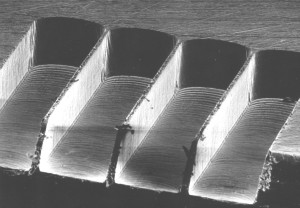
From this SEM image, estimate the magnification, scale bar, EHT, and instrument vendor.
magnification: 0.2 K X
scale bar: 100000 nm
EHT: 15 kV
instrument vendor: JEOL